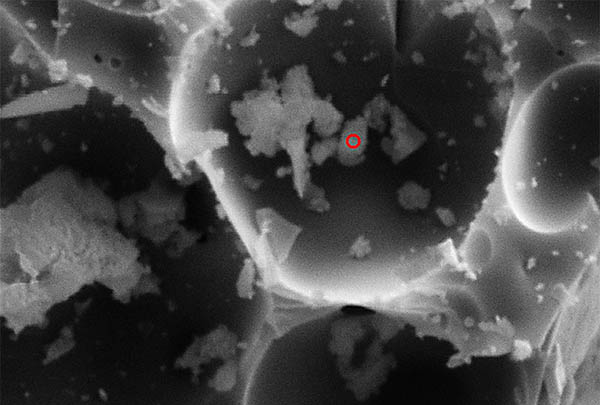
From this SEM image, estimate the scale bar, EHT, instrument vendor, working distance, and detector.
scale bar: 2000 nm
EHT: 20 kV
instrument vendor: Zeiss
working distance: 22 mm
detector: SE1